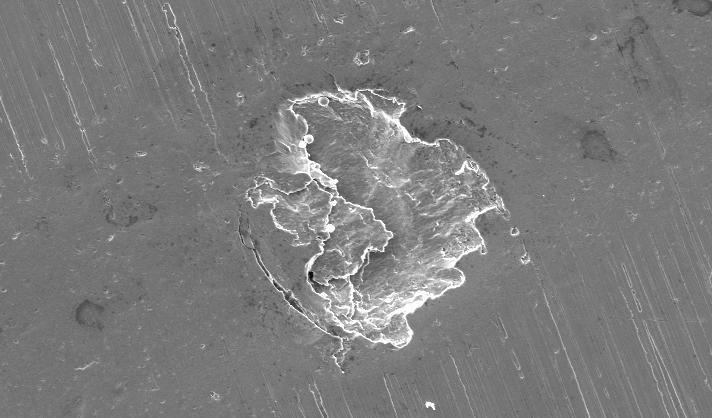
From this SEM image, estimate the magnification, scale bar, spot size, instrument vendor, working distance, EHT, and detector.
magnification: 0.2 K X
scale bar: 100000 nm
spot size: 5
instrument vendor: FEI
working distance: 13.7 mm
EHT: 20 kV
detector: SE